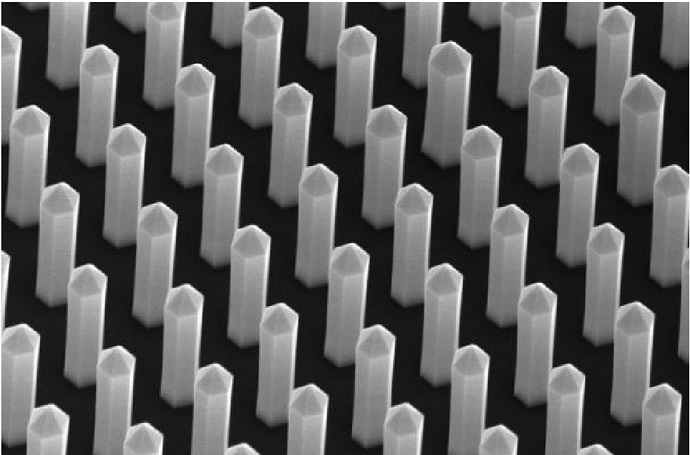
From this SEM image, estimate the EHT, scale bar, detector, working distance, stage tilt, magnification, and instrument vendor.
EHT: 10 kV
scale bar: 1000 nm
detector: InLens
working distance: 6.4 mm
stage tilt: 30°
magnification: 40 K X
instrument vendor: Zeiss